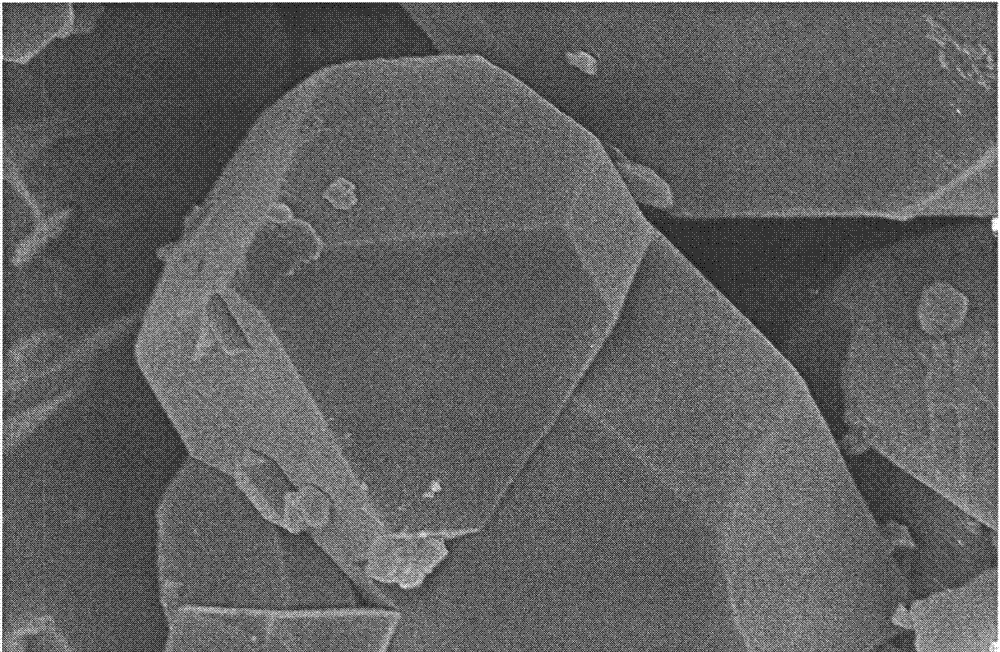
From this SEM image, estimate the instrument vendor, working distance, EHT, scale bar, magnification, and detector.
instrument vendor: Hitachi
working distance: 6.7 mm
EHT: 5 kV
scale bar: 3000 nm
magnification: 15 K X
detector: SE(M)